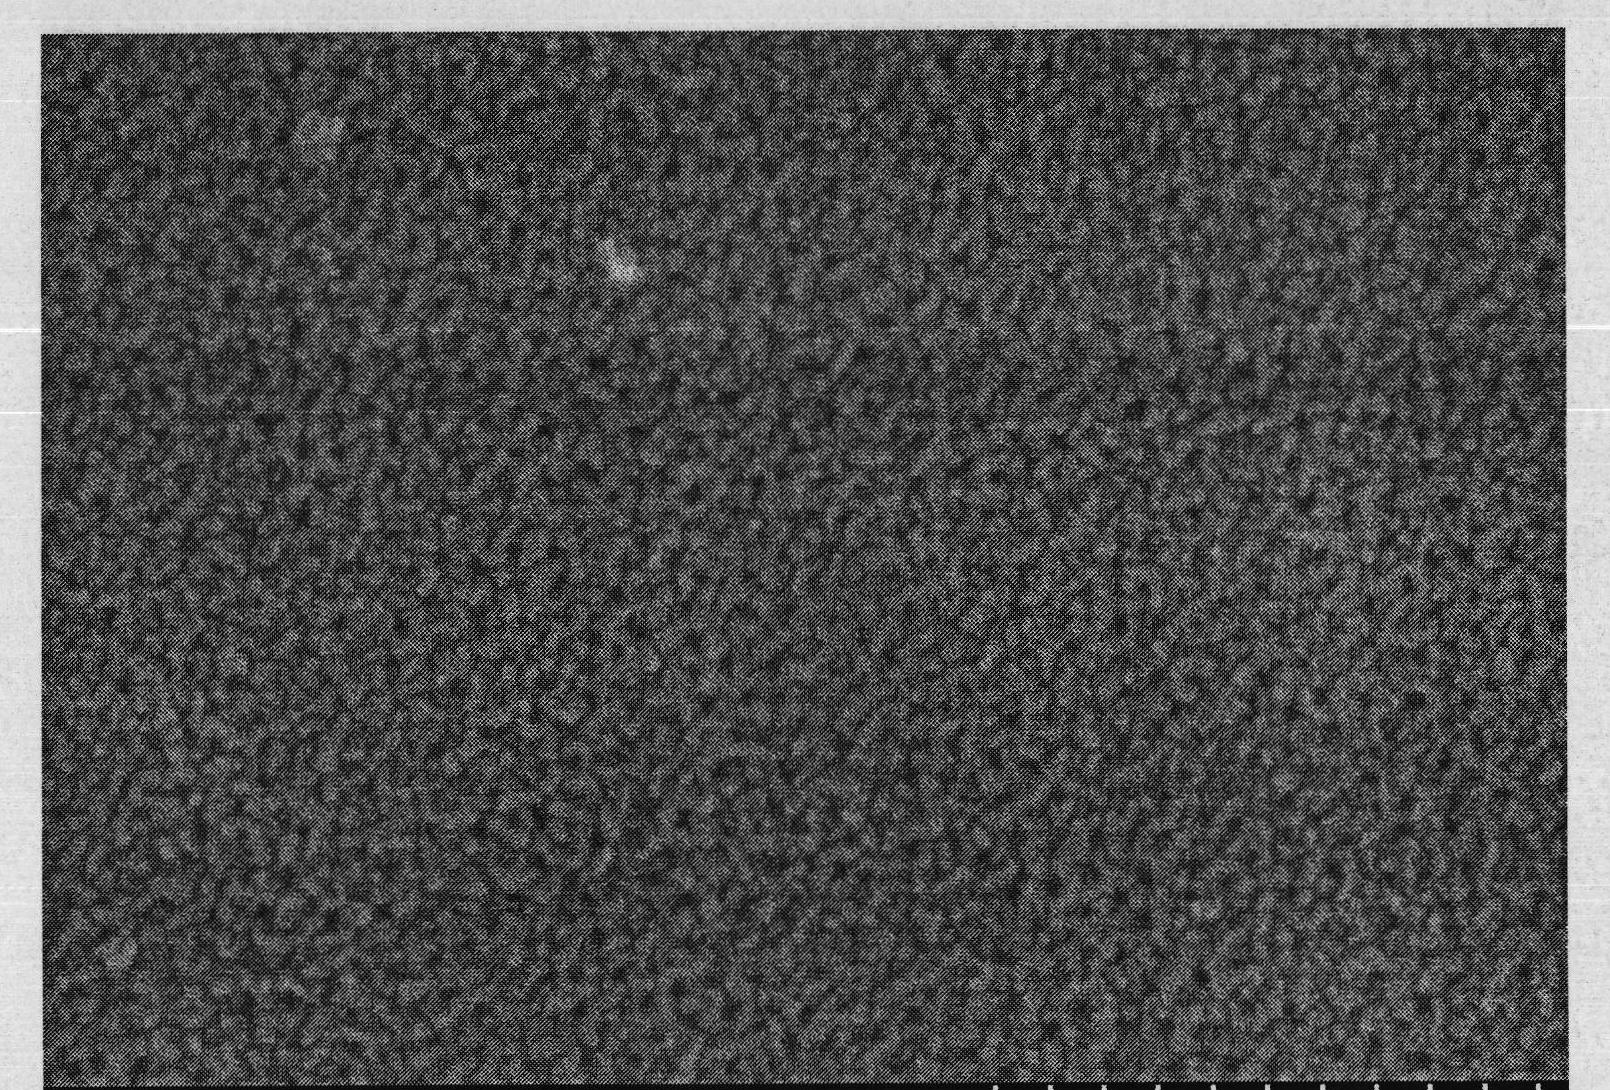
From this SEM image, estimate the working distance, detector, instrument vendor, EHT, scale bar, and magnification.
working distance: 13.3 mm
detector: SE(M)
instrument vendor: Hitachi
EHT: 10 kV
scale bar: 300 nm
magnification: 150 K X